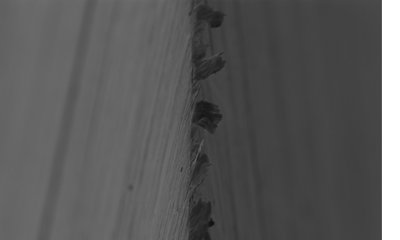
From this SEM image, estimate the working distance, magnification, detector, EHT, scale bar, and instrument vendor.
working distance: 6.5 mm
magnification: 5 K X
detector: SE2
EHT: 5 kV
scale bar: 1000 nm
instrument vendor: Zeiss